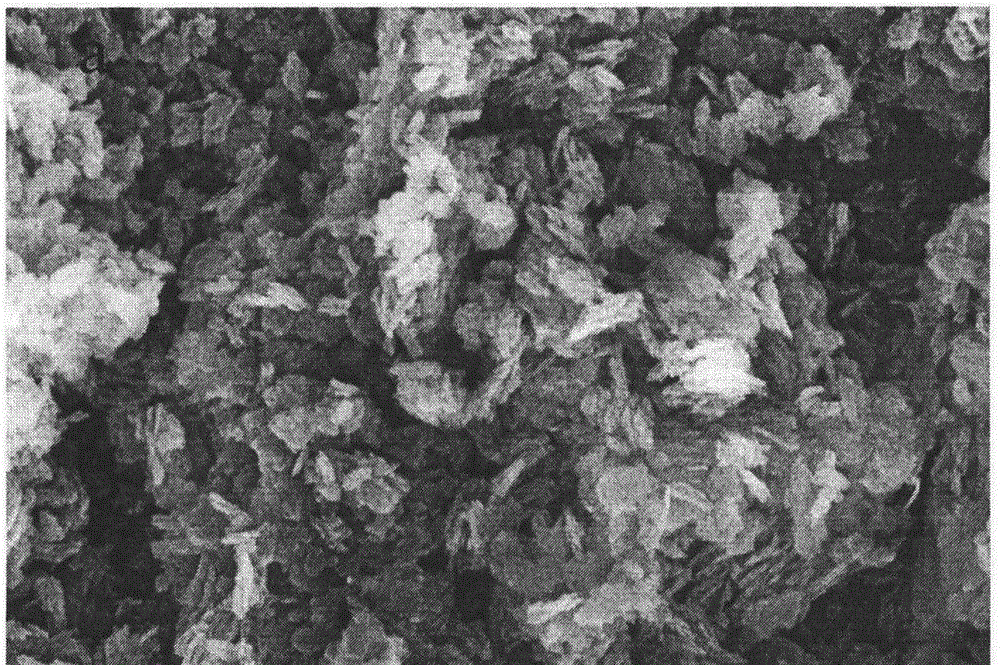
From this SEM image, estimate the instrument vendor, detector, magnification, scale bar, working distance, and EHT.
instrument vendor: Zeiss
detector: InlensDuo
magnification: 50 K X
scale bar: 100 nm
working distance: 11.8 mm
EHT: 10 kV